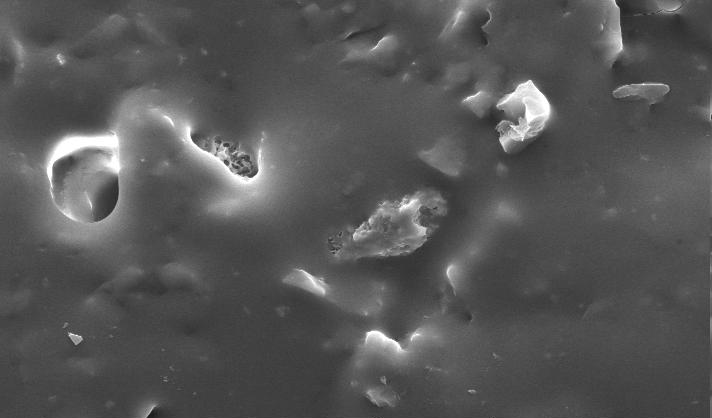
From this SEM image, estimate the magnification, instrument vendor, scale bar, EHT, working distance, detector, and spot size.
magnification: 1 K X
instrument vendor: FEI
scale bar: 20000 nm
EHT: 25 kV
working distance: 10.1 mm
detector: SE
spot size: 4